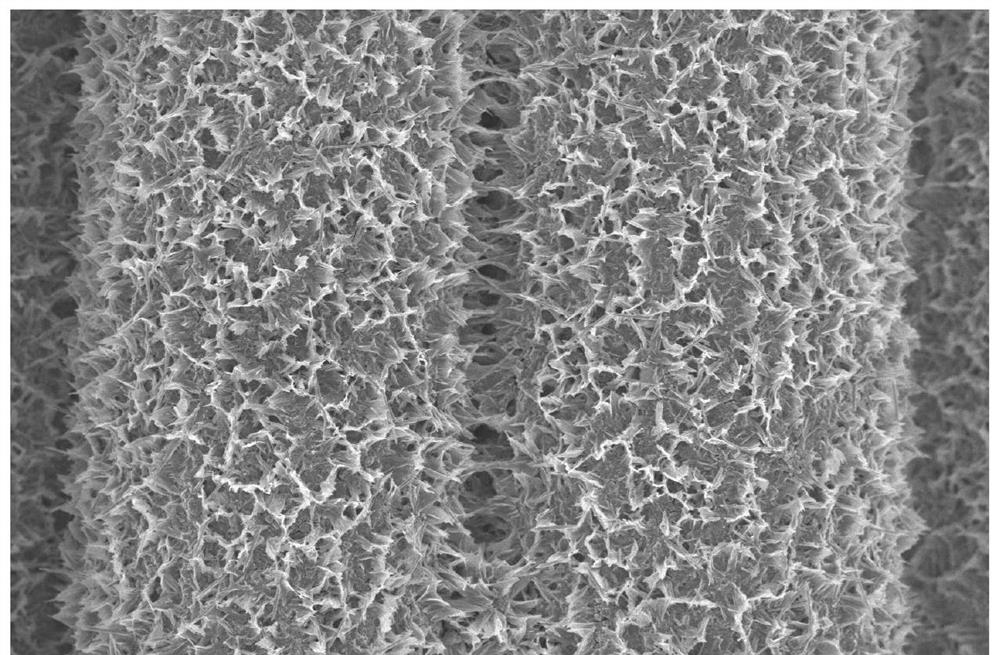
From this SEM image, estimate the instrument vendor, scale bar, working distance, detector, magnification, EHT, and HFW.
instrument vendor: FEI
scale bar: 4000 nm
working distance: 4.7 mm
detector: TLD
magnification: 20 K X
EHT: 2 kV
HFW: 20.7 µm